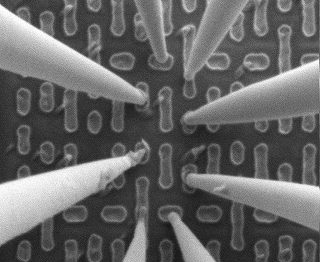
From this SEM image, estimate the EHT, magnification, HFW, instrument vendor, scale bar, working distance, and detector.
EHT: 2 kV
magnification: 85.931 K X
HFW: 3.15 µm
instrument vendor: FEI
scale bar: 1000 nm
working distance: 8 mm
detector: ETD